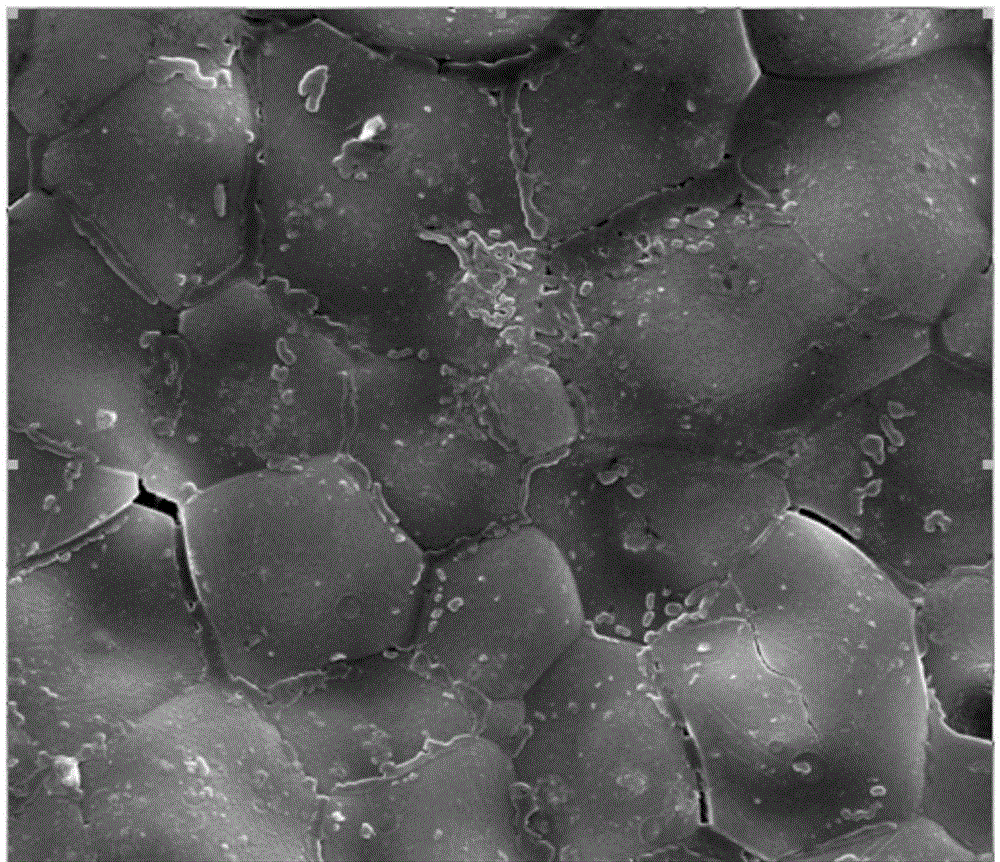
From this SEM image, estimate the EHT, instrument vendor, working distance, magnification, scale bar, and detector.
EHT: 20 kV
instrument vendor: FEI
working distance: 9.3 mm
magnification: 10 K X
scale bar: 10000 nm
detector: SE